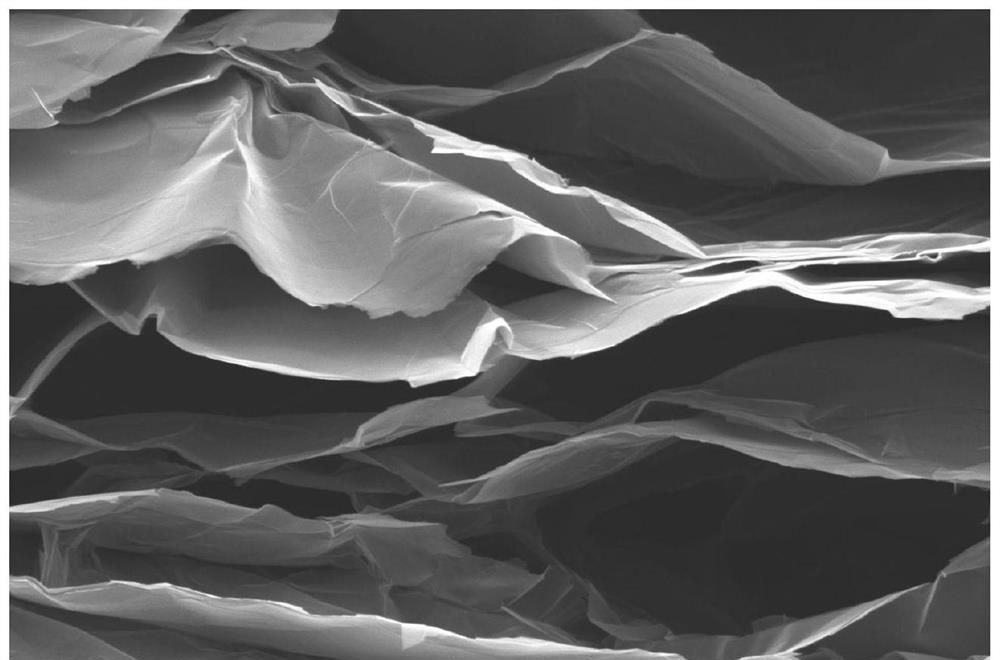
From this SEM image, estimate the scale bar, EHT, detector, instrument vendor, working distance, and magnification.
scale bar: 10000 nm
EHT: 5 kV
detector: ETD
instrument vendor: FEI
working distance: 6.5 mm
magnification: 5 K X